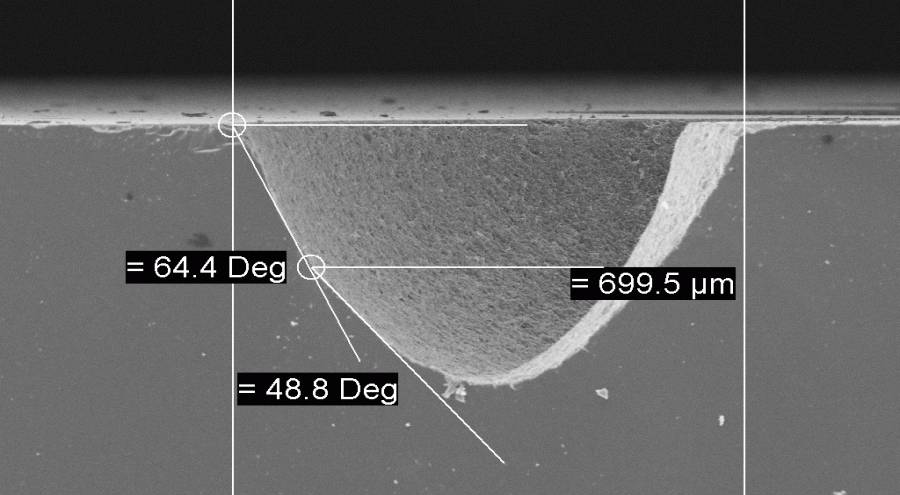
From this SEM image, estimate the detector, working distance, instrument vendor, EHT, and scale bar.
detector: SE1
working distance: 42 mm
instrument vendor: Zeiss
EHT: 20 kV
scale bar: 200000 nm